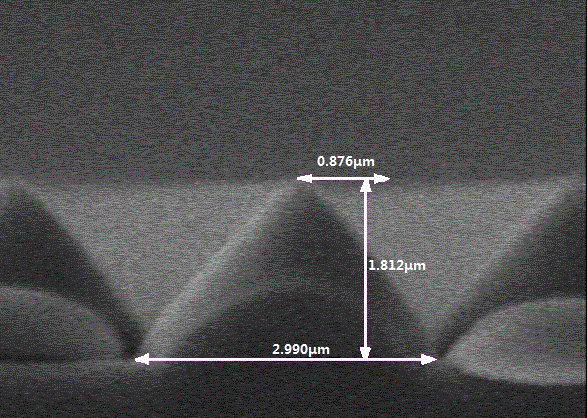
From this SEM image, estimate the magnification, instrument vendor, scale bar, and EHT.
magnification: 20 K X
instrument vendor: JEOL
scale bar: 1500 nm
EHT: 10 kV